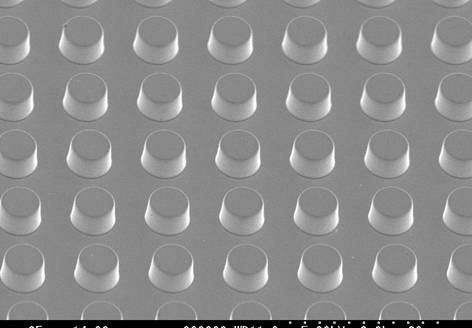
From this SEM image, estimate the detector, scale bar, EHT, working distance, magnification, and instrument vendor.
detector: SE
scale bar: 20000 nm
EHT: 5 kV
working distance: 11 mm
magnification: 2 K X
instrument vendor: Hitachi